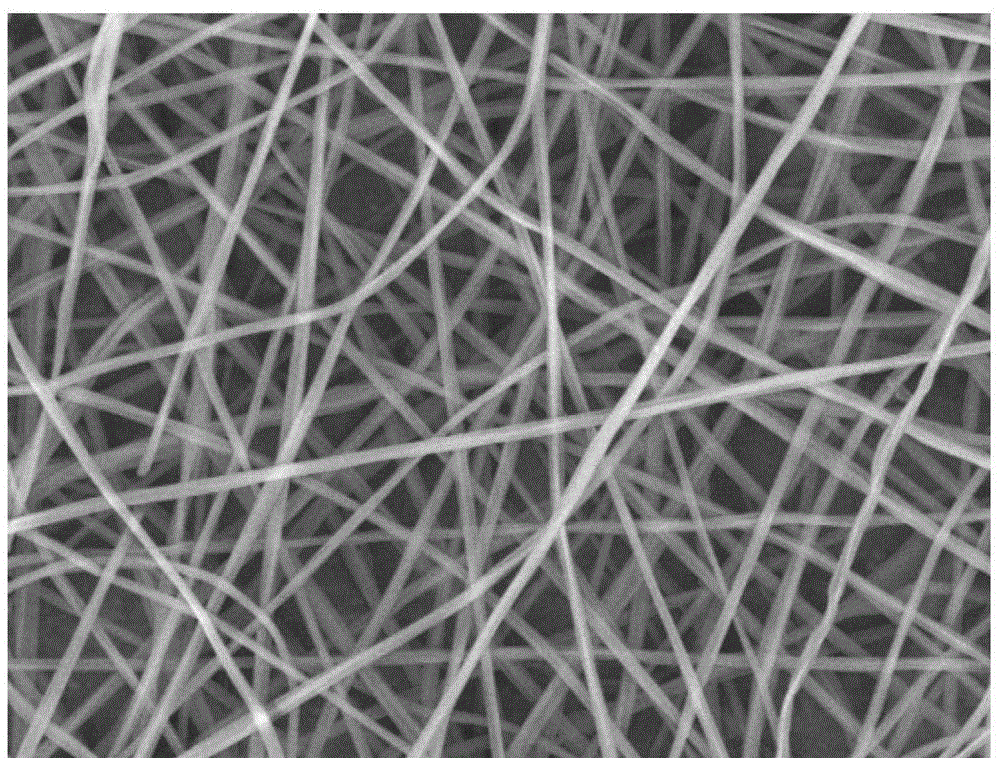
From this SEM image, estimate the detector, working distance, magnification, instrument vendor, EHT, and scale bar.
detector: LEI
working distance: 9.2 mm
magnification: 3 K X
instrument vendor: JEOL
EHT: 10 kV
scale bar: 1000 nm